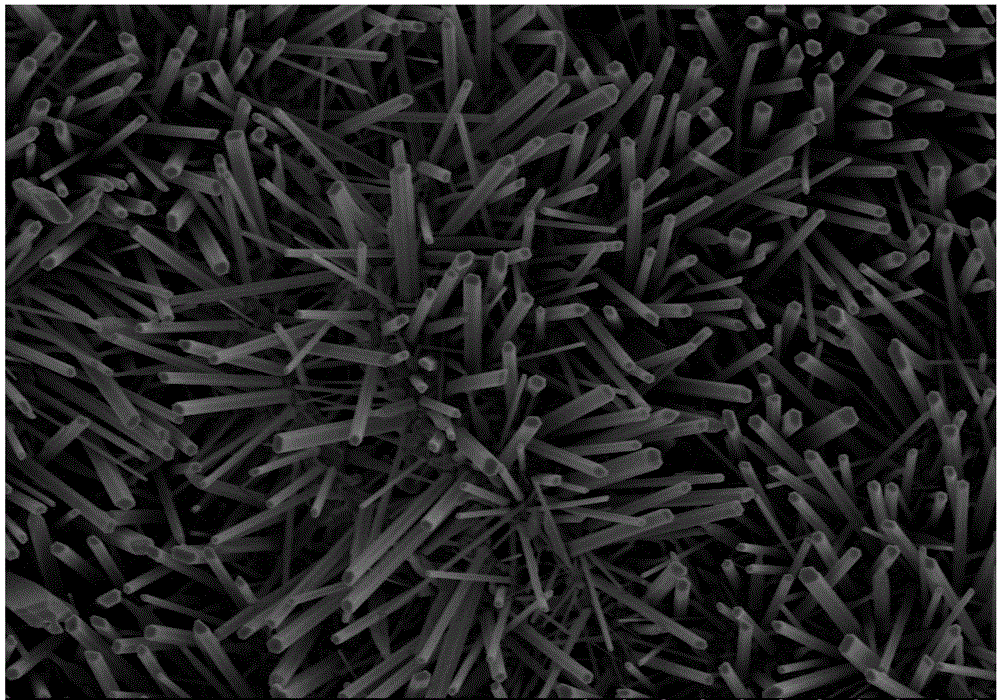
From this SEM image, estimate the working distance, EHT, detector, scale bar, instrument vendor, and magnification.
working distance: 9.4 mm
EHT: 5 kV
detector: SE(M)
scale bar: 10000 nm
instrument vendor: Hitachi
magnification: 5 K X